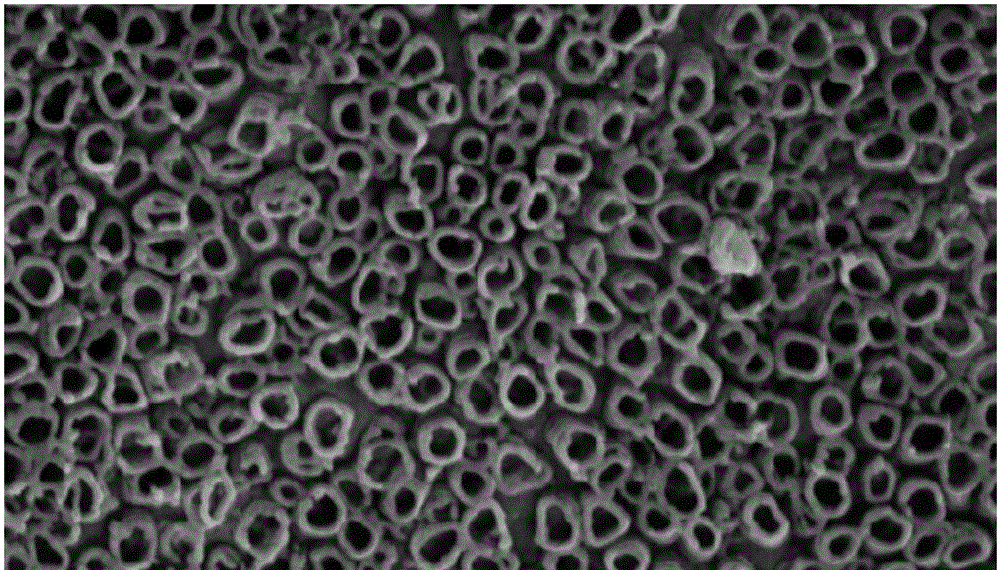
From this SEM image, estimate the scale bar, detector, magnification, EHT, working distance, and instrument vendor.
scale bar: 200 nm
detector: SE2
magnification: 100 K X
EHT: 15 kV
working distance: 11 mm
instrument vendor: Zeiss